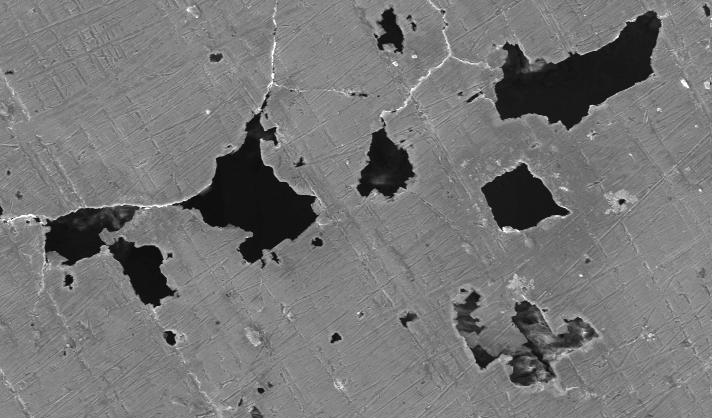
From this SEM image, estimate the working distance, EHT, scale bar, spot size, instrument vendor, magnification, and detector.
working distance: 26.2 mm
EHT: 20 kV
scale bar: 100000 nm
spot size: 4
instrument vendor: FEI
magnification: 0.5 K X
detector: SE